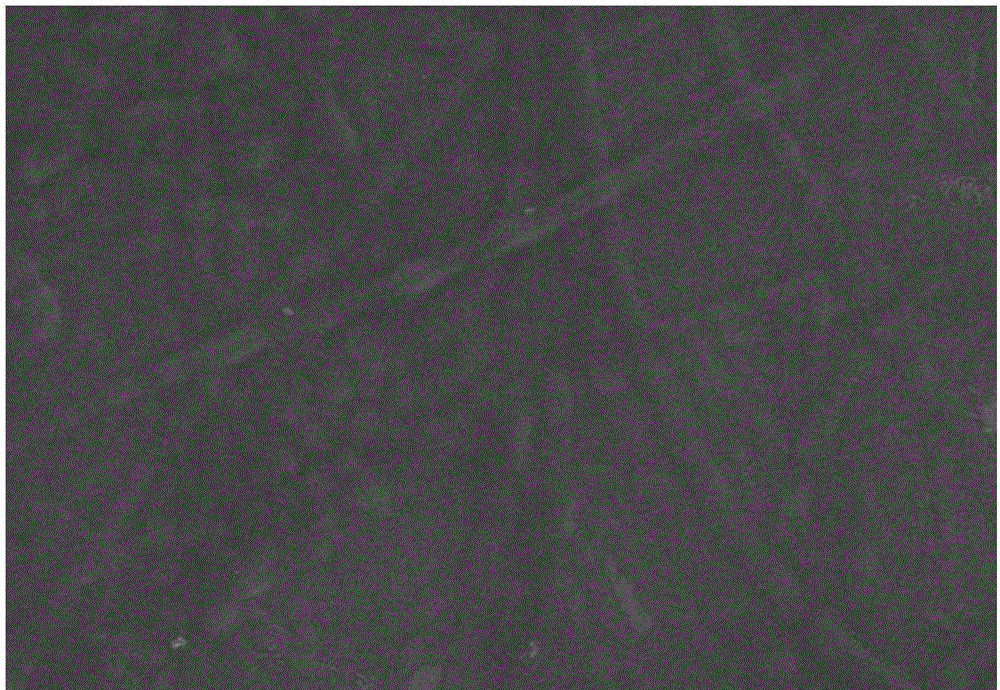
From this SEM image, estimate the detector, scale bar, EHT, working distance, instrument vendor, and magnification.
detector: SE(M)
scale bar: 5000 nm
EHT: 5 kV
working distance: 8.2 mm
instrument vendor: Hitachi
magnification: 10 K X